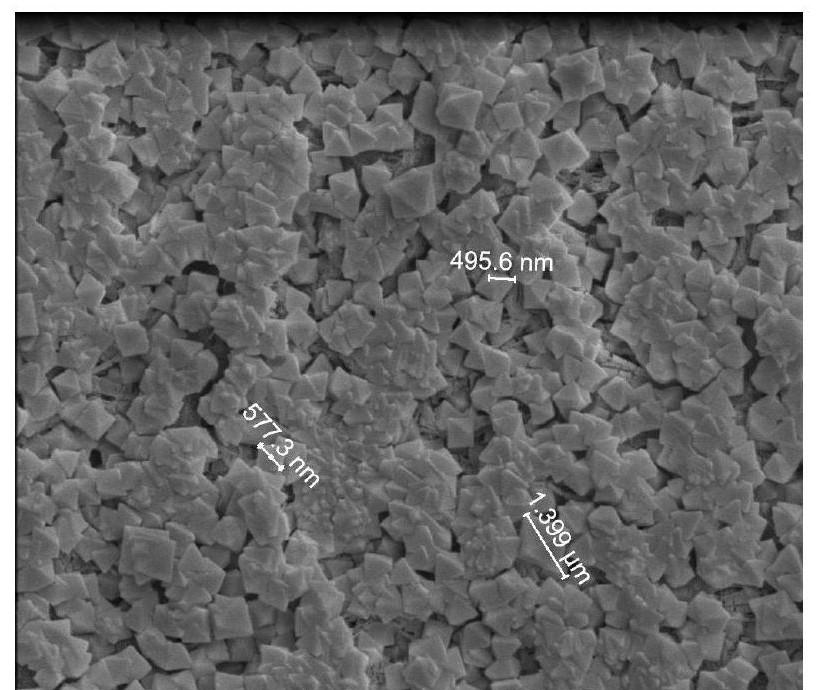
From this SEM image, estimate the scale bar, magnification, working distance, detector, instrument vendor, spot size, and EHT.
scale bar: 4000 nm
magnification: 20 K X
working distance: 7.9 mm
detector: ETD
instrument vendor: FEI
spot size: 3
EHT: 5 kV